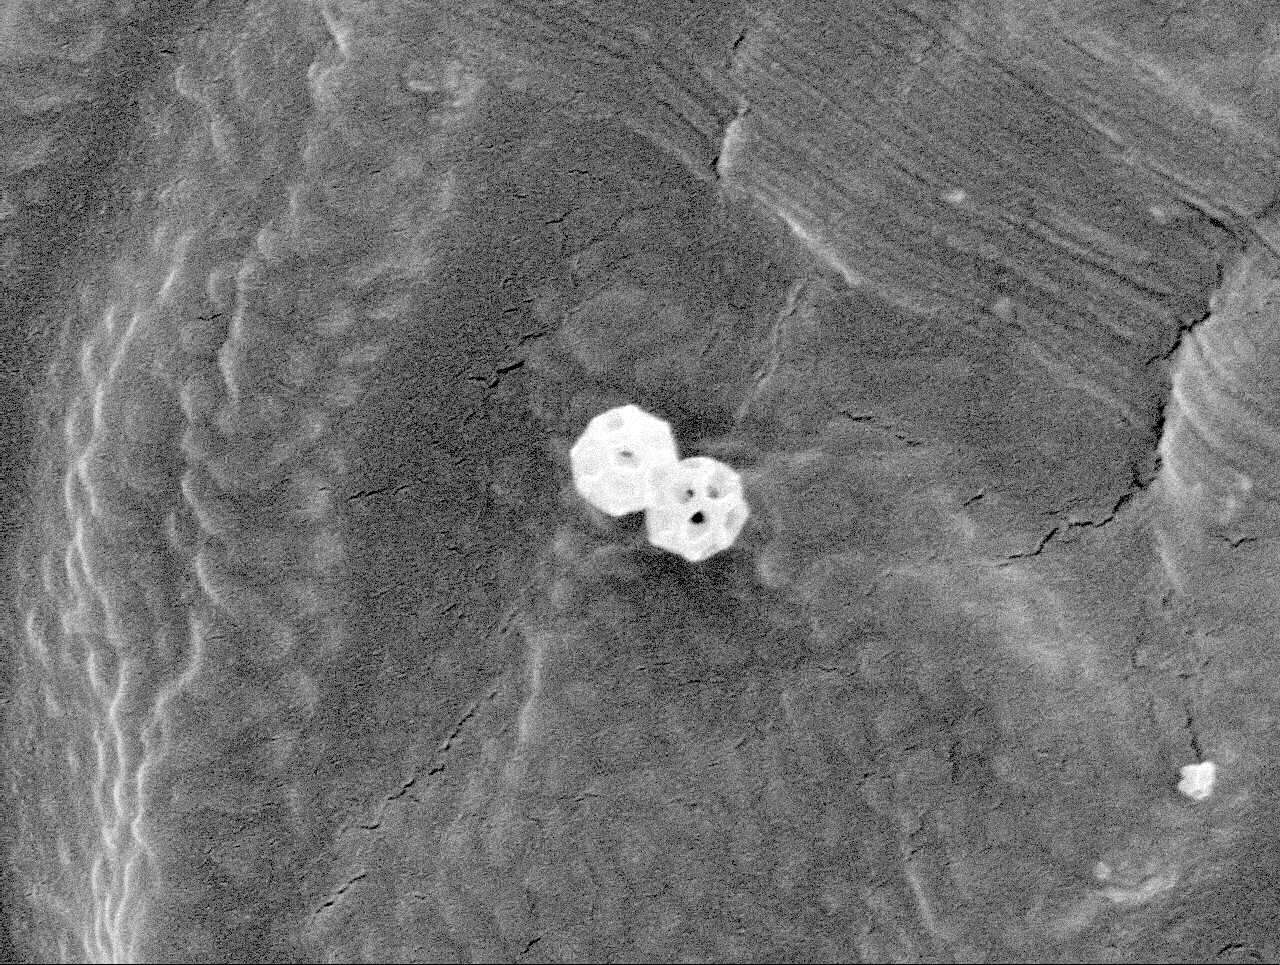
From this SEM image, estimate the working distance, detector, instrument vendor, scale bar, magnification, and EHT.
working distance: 10.1 mm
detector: SEI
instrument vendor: JEOL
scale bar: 1000 nm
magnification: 25 K X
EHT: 5 kV